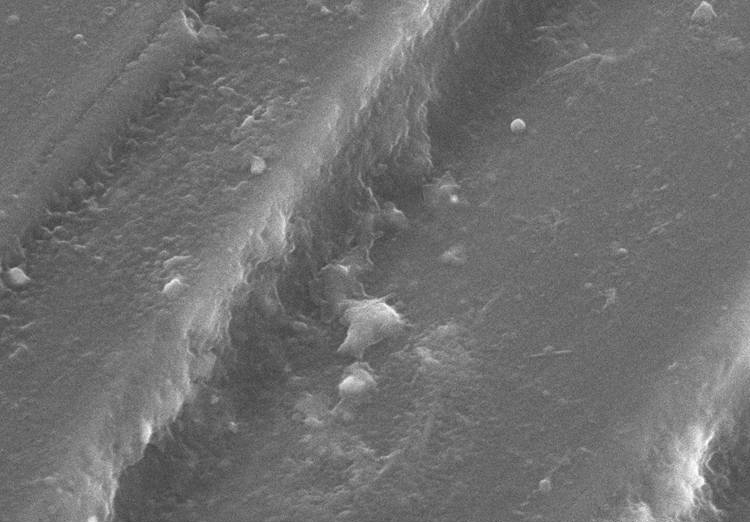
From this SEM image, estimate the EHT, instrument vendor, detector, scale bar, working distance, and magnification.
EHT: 15 kV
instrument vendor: Hitachi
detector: SE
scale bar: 10000 nm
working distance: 12.7 mm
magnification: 4 K X